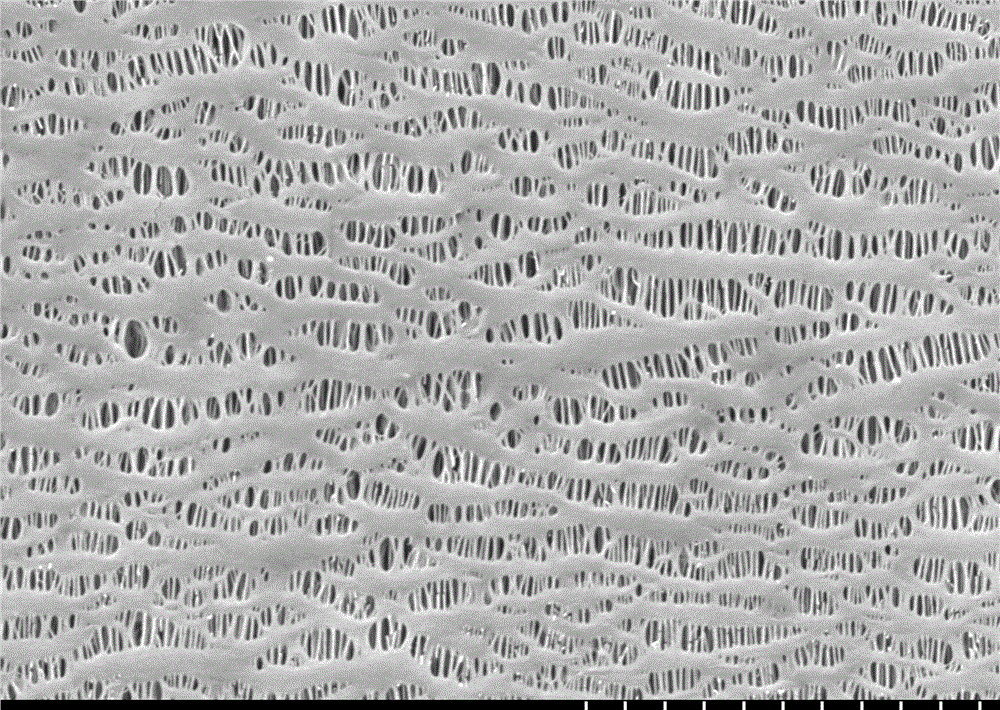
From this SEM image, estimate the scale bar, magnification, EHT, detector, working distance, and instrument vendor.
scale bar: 5000 nm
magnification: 10 K X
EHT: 5 kV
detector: SE(M,LA0)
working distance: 8.9 mm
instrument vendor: Hitachi